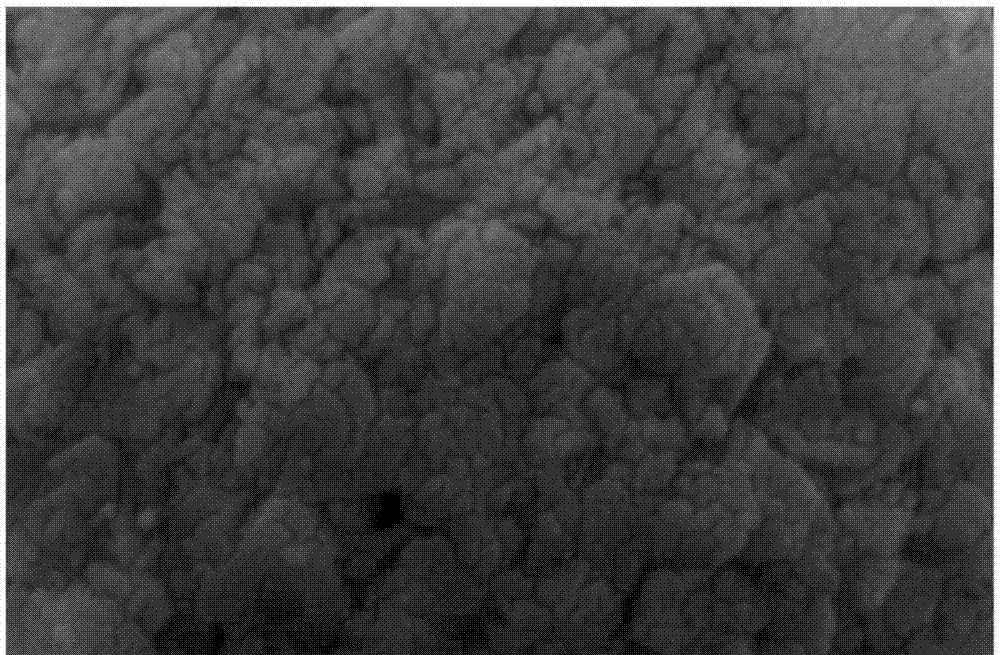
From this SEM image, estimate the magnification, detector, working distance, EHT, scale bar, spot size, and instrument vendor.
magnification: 50 K X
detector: SE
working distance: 10.3 mm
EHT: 10 kV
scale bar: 1000 nm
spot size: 4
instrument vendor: FEI